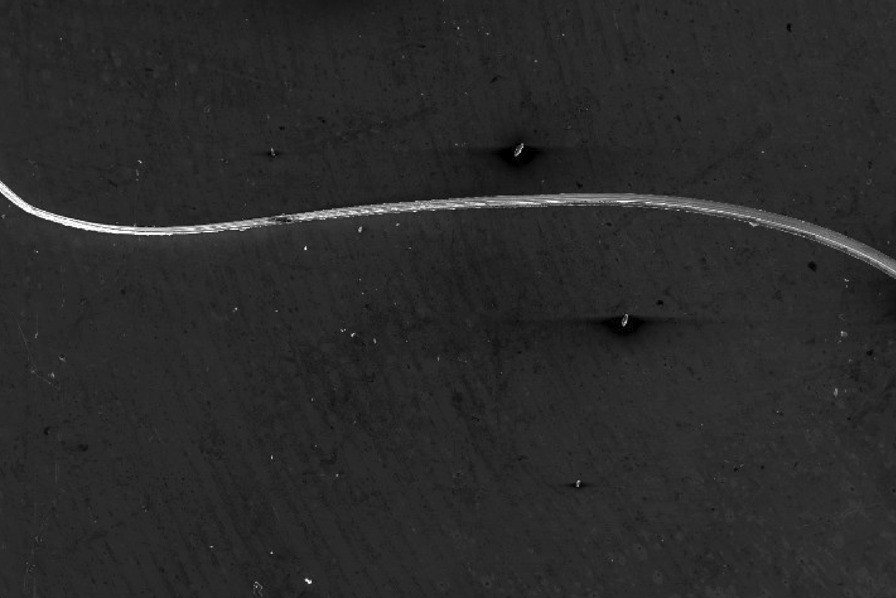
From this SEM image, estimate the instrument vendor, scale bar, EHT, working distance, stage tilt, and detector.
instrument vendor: FEI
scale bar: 1000000 nm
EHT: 20 kV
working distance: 14.7 mm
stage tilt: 0°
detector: SE